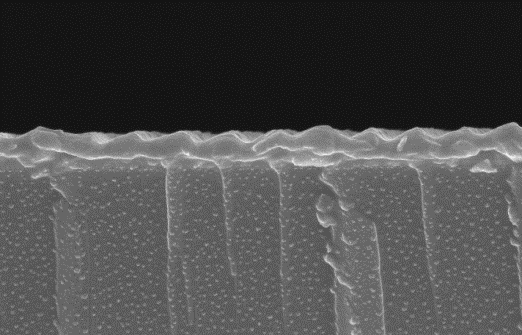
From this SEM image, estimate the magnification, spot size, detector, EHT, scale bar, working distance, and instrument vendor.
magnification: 50 K X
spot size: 3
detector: TLD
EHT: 10 kV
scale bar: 1000 nm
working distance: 5.2 mm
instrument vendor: FEI